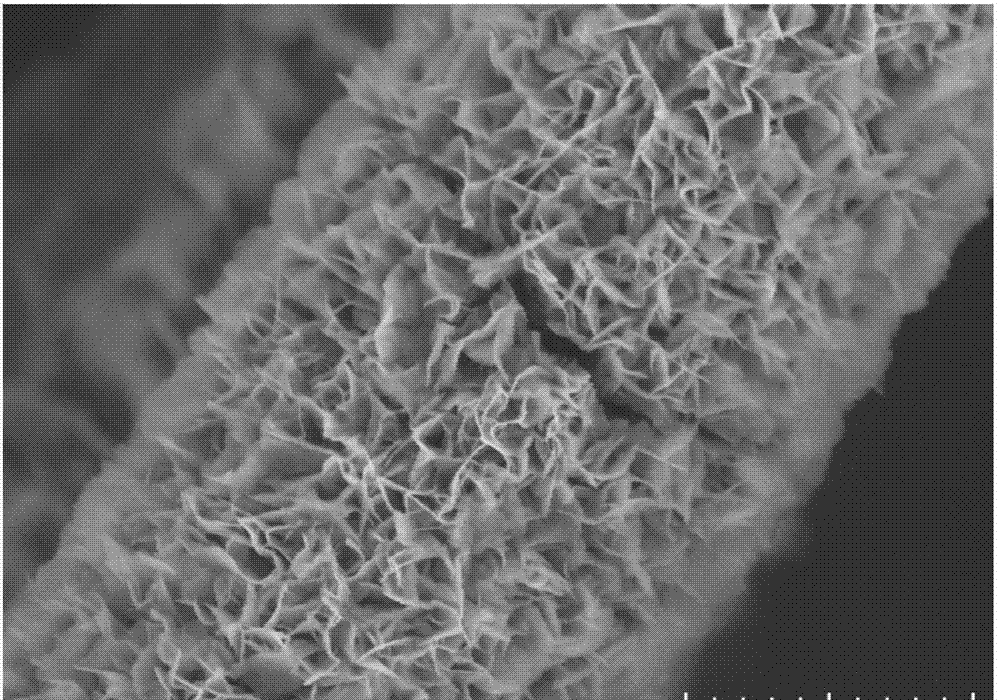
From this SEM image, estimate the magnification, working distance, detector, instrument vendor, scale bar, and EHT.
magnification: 10 K X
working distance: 10.2 mm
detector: SE(UL)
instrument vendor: Hitachi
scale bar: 5000 nm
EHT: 5 kV